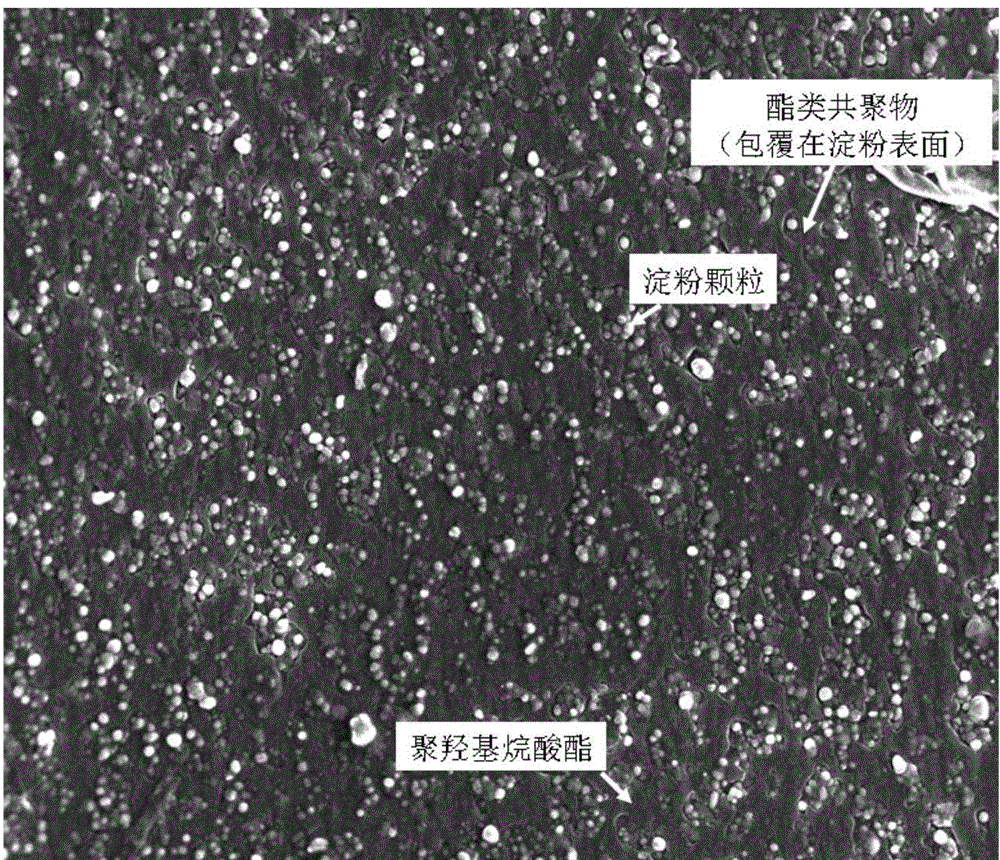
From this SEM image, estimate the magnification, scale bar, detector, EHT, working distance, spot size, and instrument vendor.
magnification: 2 K X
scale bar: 50000 nm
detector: SE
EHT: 10 kV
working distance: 10 mm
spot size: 5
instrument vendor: FEI